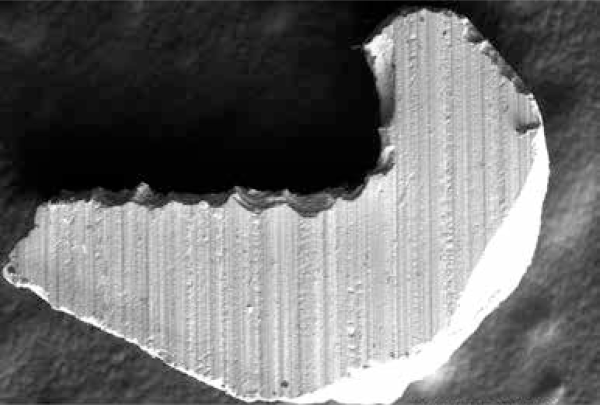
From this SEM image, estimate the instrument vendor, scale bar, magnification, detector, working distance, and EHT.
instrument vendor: JEOL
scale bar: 500000 nm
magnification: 0.06 K X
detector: BSE1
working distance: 14.1 mm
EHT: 20 kV